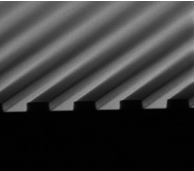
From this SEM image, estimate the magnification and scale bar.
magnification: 20 K X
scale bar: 3000 nm